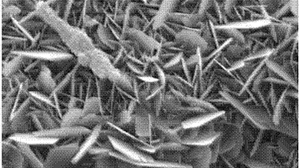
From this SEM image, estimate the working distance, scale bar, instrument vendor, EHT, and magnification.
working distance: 12 mm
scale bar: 1000 nm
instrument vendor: Zeiss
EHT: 15 kV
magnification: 10 K X